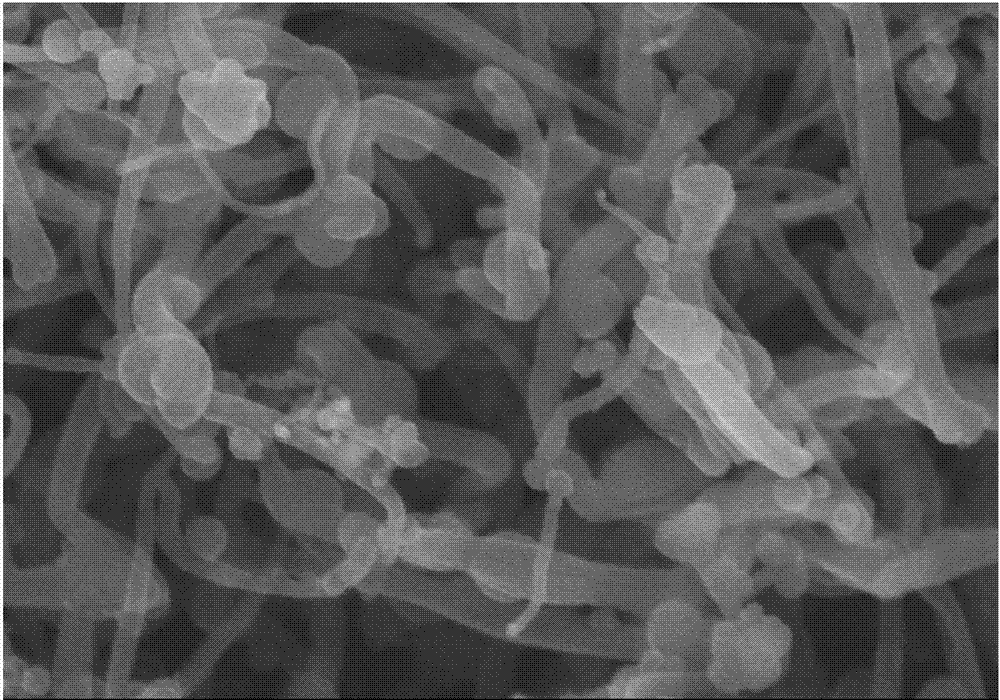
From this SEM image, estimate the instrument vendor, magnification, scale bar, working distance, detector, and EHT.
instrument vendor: Hitachi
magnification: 100 K X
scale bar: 500 nm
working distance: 8.5 mm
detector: SE(M)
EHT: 15 kV